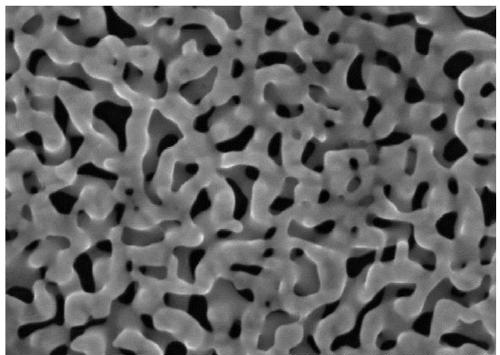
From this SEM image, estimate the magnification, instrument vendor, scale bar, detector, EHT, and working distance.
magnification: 100 K X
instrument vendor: Hitachi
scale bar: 500 nm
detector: SE(U)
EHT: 15 kV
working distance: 10.4 mm